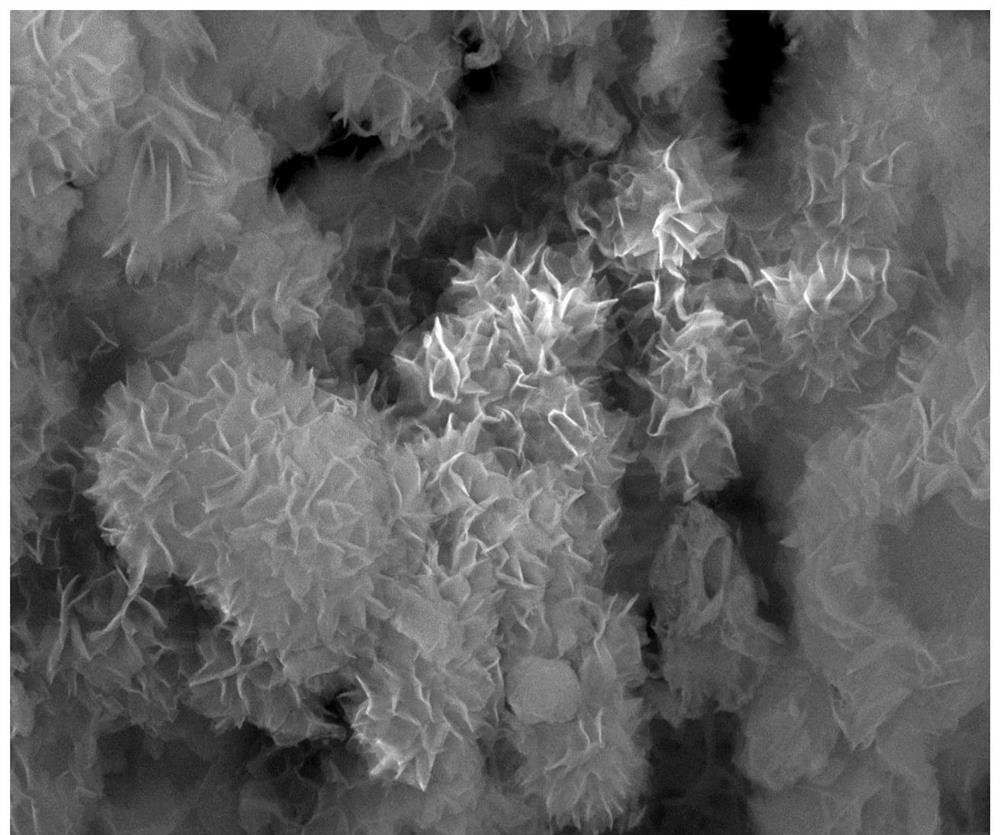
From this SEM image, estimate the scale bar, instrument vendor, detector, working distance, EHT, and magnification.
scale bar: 1000 nm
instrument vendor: JEOL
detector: SEI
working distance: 10.2 mm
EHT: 15 kV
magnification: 15 K X